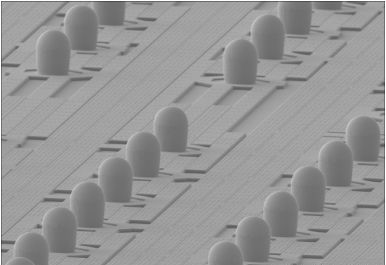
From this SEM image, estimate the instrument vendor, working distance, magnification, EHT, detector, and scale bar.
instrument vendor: Hitachi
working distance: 7.5 mm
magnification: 0.35 K X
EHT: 15 kV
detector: SE(M)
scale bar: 100000 nm